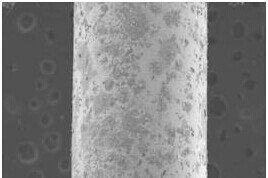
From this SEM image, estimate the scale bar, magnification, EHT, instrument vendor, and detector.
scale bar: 500000 nm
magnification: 0.05 K X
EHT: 15 kV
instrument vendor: JEOL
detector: SEI